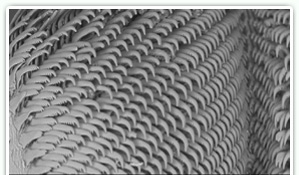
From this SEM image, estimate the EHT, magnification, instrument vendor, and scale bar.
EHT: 15 kV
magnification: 0.15 K X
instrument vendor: Hitachi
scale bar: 300000 nm